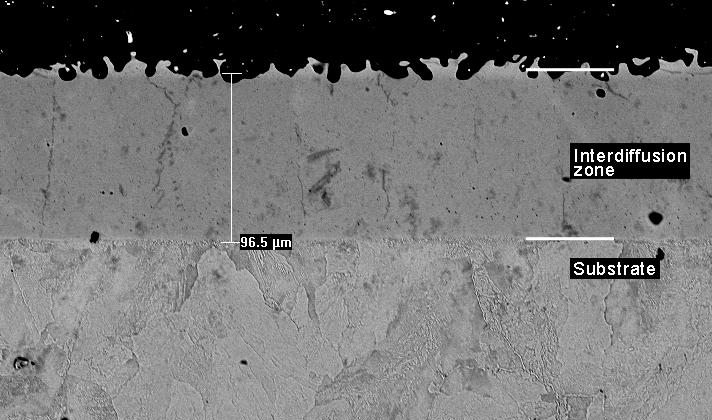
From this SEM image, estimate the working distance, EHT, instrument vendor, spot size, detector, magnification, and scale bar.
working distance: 11.9 mm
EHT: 20 kV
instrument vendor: FEI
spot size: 3.9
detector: BSE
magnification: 0.25 K X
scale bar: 100000 nm